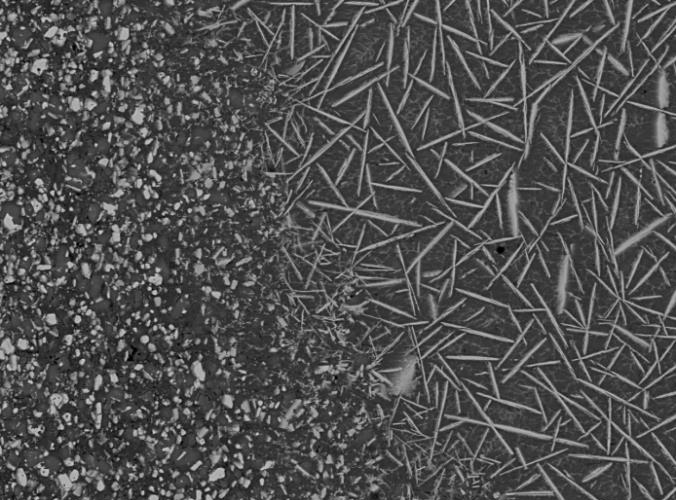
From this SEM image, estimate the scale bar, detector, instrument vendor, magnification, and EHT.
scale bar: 10000 nm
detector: COMPO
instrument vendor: JEOL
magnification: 0.5 K X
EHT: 15 kV